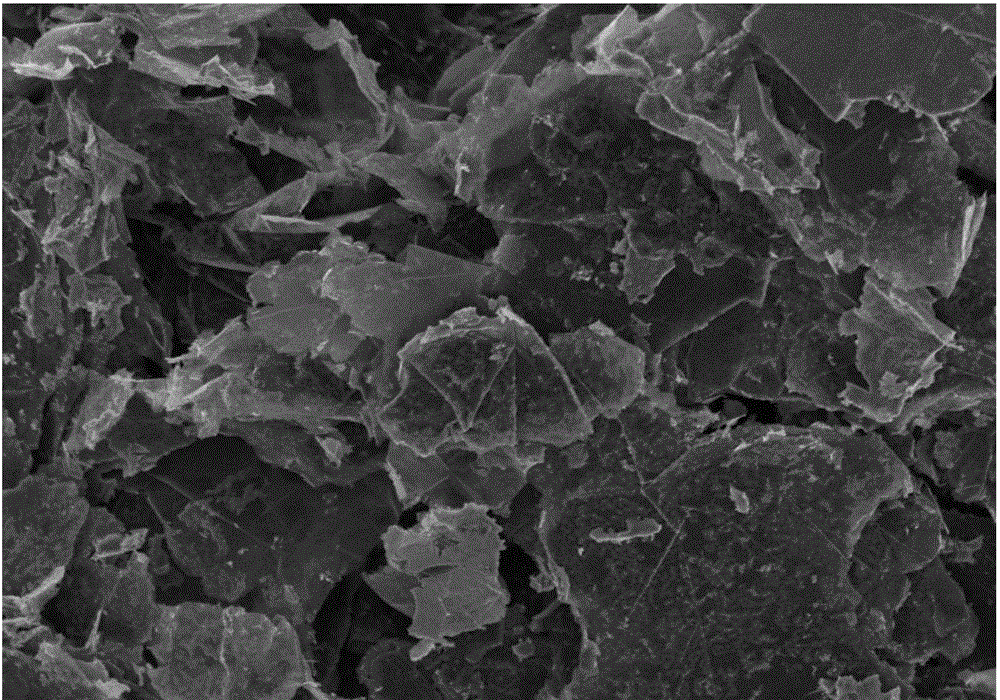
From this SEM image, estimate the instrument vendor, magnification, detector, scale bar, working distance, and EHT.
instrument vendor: Hitachi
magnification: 5 K X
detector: SE(M)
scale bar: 10000 nm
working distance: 7.8 mm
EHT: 3 kV